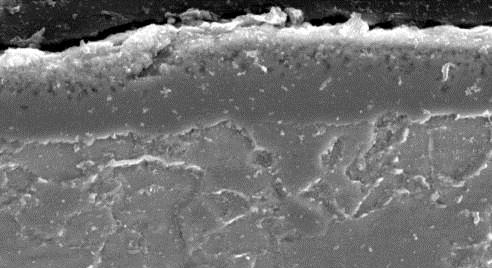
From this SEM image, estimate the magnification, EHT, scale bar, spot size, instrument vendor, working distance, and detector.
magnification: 3.5 K X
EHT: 20 kV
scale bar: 10000 nm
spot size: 3.5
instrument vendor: FEI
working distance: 10.8 mm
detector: SE